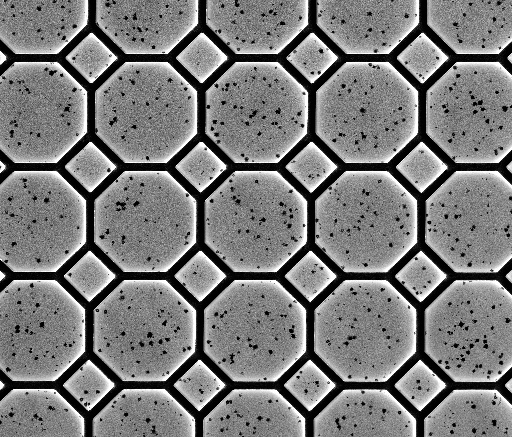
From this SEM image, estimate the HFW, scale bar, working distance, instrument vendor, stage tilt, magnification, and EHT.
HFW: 256 µm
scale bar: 50000 nm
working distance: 9.1 mm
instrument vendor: FEI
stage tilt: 0°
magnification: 0.5 K X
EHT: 3.2 kV